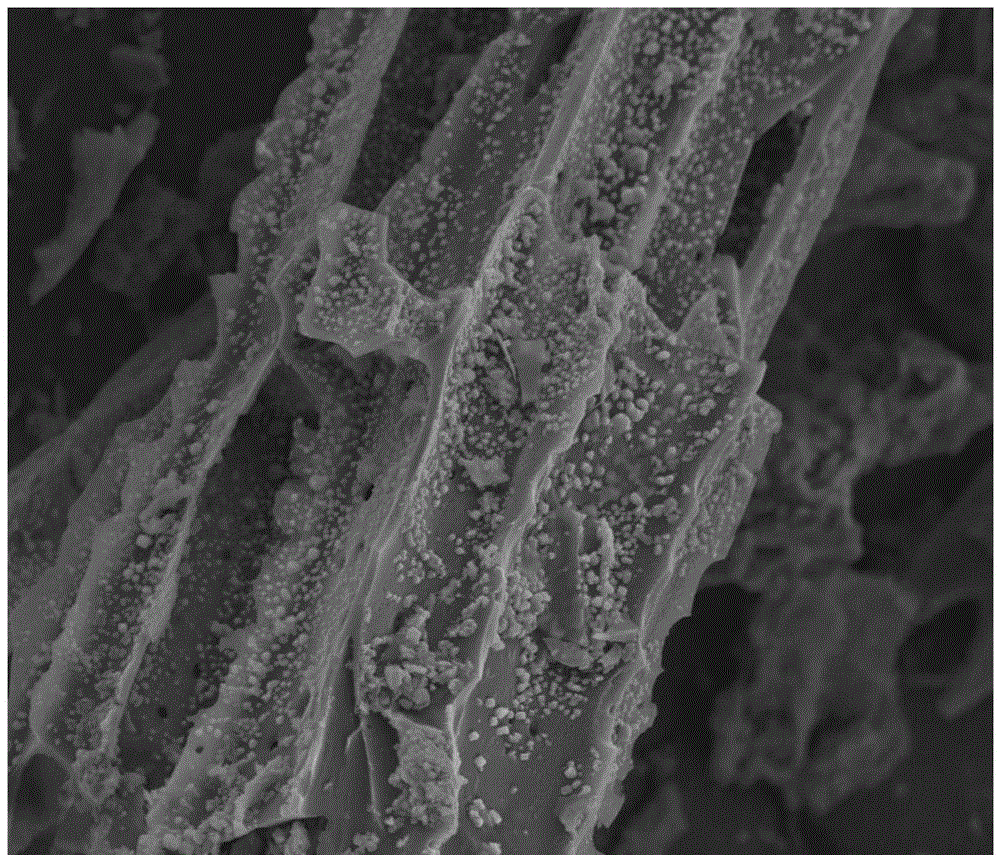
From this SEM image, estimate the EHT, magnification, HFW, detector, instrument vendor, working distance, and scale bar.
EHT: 10 kV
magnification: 4 K X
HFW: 74.6 µm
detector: ETD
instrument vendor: FEI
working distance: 8.2 mm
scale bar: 30000 nm